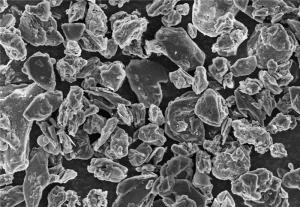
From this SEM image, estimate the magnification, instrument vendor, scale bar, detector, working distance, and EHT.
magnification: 1 K X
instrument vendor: Hitachi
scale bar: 50000 nm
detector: SE(U)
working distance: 8 mm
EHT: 10 kV